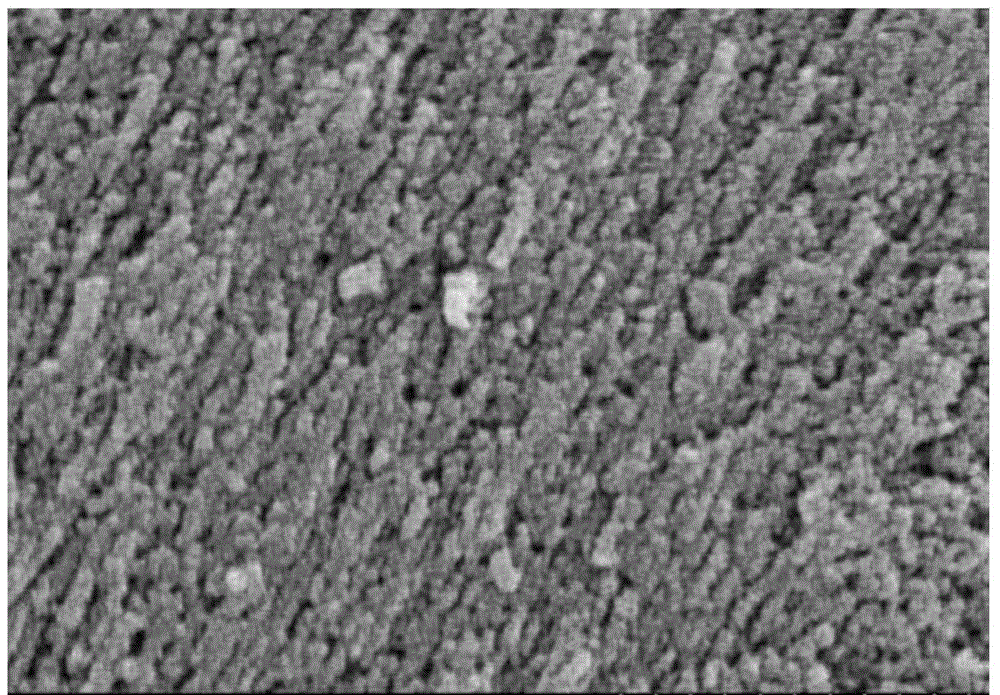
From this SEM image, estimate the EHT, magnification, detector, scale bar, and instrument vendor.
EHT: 5 kV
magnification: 90 K X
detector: SE(M)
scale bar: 500 nm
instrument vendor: Hitachi